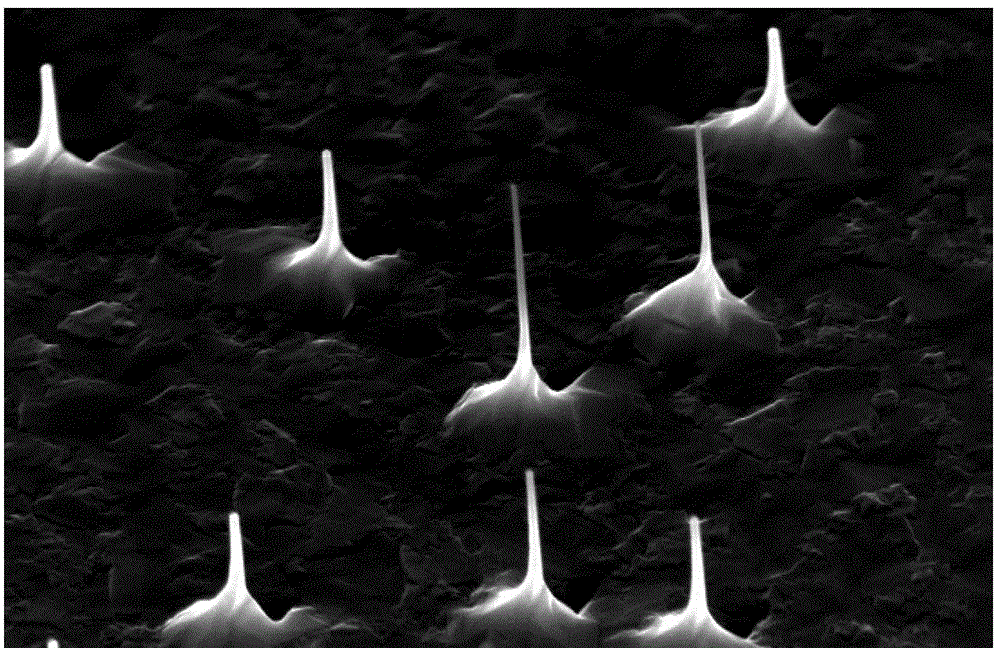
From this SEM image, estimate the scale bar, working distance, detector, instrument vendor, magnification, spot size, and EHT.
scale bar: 2000 nm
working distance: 6.2 mm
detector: TLD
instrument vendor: FEI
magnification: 40 K X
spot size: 4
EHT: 15 kV